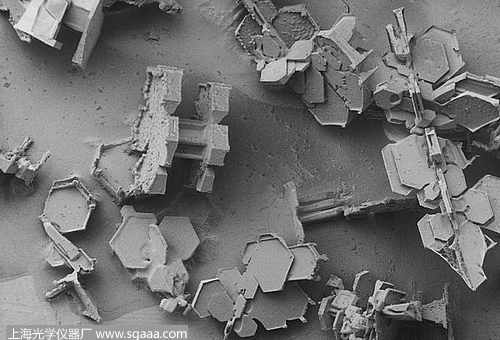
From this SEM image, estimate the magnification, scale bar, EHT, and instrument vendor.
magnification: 0.03 K X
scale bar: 1000000 nm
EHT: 2 kV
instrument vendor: JEOL